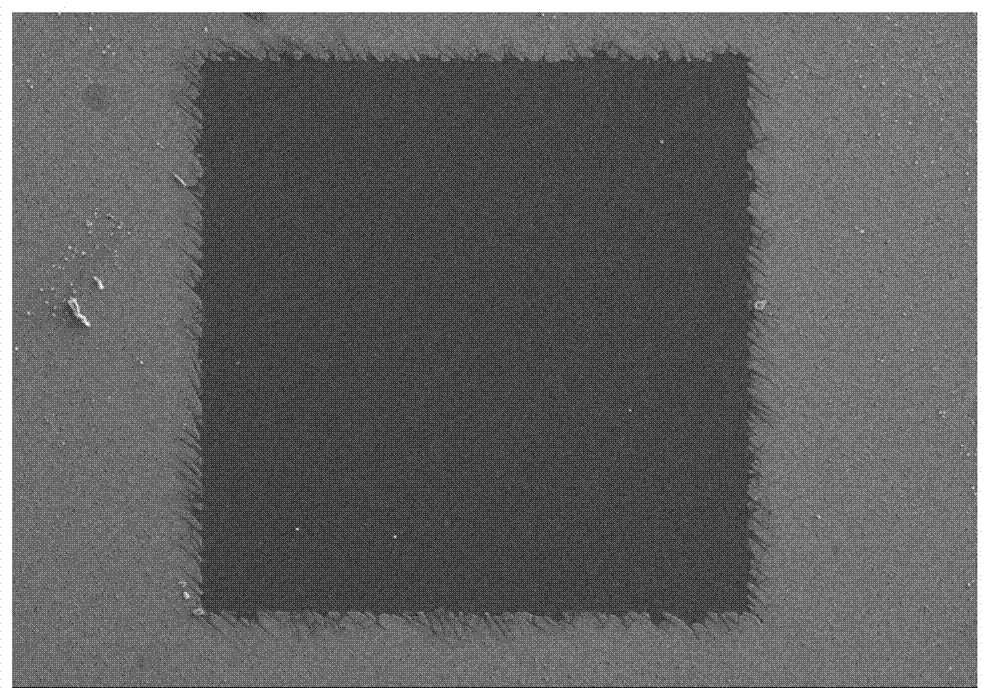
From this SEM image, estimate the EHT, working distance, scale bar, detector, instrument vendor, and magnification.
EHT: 12 kV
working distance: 5.7 mm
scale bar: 400000 nm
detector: SE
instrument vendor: Hitachi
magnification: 0.12 K X